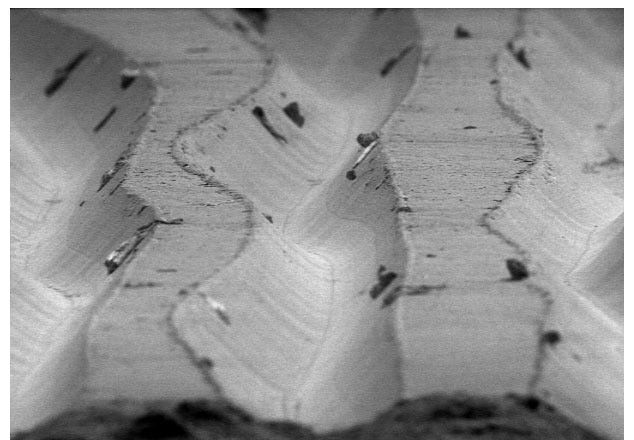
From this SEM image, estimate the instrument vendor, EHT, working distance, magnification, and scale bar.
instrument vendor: JEOL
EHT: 5 kV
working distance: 27 mm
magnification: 0.5 K X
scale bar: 50000 nm